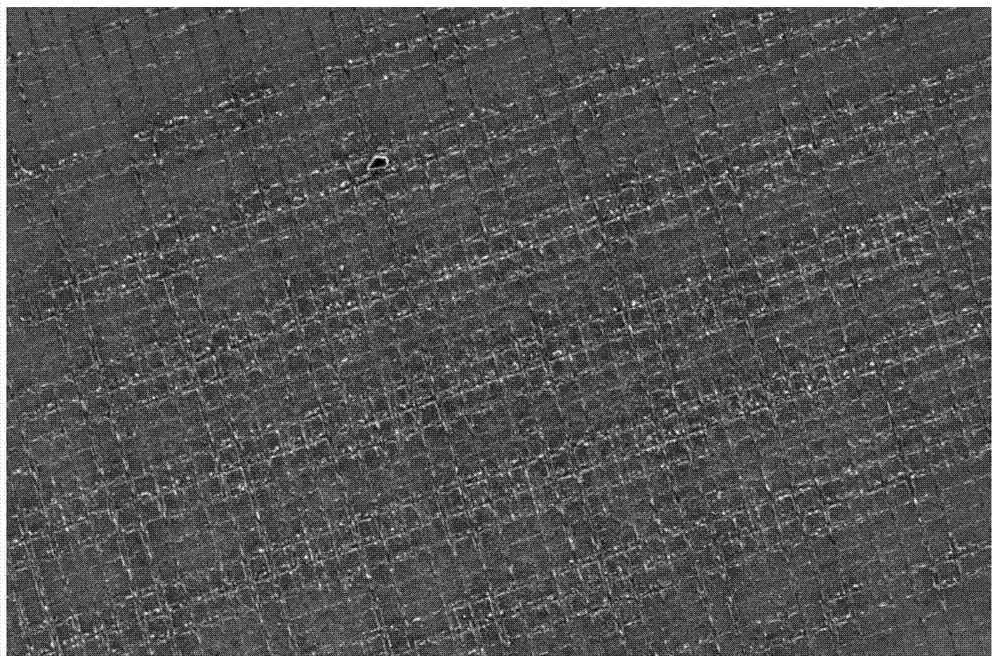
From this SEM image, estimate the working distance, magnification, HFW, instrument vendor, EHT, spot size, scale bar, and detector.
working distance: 7.2 mm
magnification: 1.2 K X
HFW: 345 µm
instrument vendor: FEI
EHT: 7 kV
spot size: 3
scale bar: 100000 nm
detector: ETD